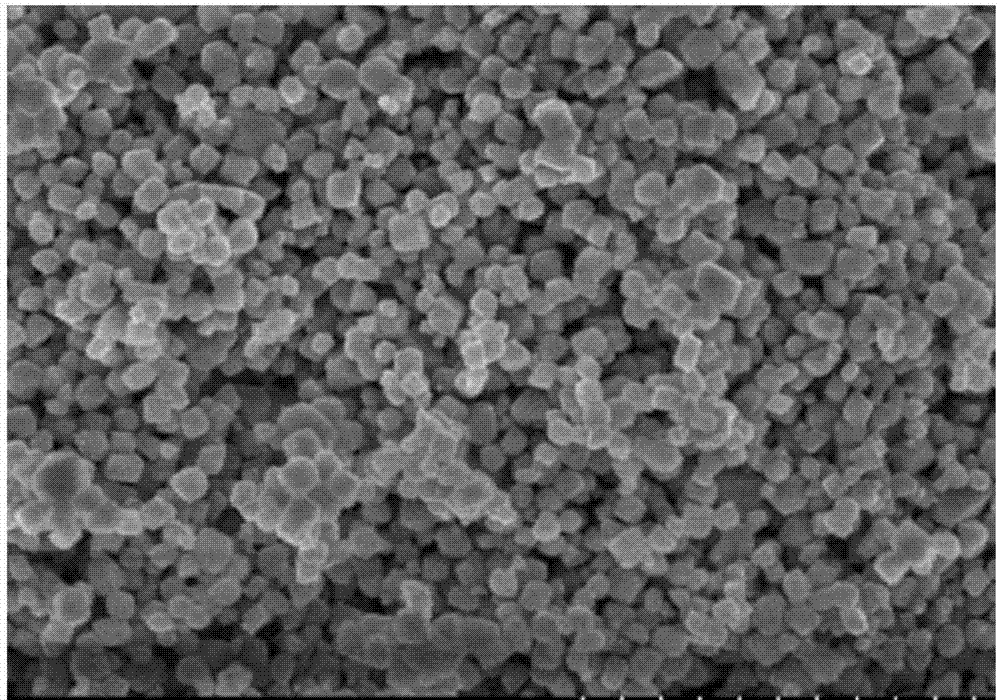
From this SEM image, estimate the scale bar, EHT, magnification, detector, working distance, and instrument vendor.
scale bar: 1000 nm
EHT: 3 kV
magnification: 50 K X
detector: SE(U)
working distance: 7.7 mm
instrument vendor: Hitachi